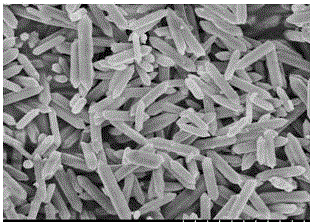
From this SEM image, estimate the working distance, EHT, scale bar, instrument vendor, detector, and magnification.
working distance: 8.9 mm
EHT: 5 kV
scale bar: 5000 nm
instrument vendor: Hitachi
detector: SE(M,LA0)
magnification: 10 K X